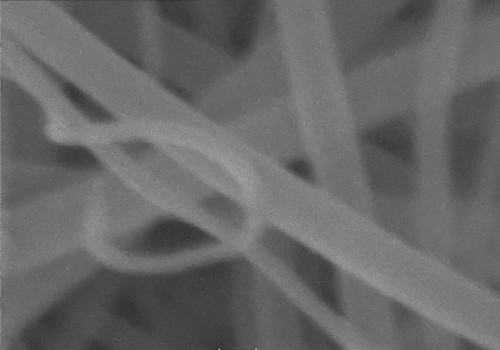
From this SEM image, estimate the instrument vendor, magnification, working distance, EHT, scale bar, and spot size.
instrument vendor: other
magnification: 150 K X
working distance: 5.9 mm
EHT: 20 kV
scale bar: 100 nm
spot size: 5